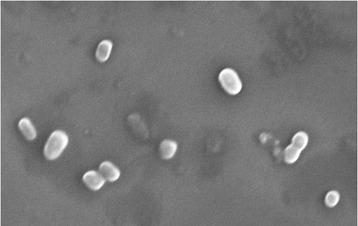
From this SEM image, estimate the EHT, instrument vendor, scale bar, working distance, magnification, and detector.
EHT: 15 kV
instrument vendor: Hitachi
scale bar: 5000 nm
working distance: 14.3 mm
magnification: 8 K X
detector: SE